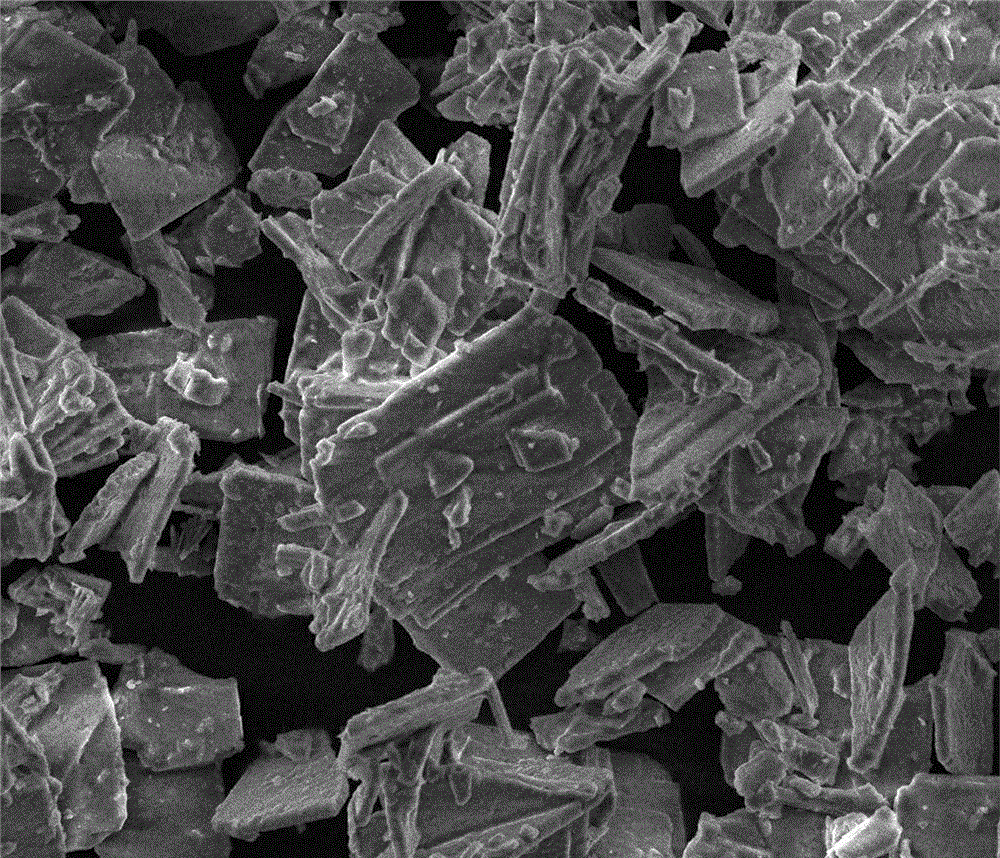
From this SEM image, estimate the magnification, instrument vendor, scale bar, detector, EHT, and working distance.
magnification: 2 K X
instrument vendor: FEI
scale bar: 50000 nm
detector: ETD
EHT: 10 kV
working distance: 6.2 mm